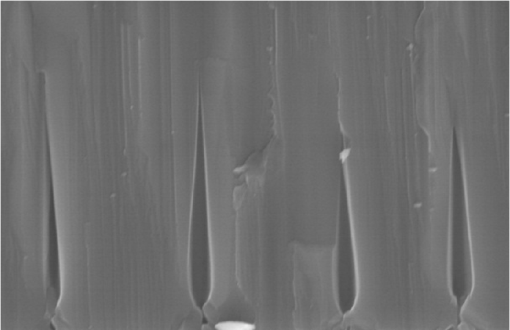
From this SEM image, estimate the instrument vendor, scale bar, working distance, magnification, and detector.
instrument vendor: Hitachi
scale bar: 2000 nm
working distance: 8 mm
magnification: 25 K X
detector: SE(M)